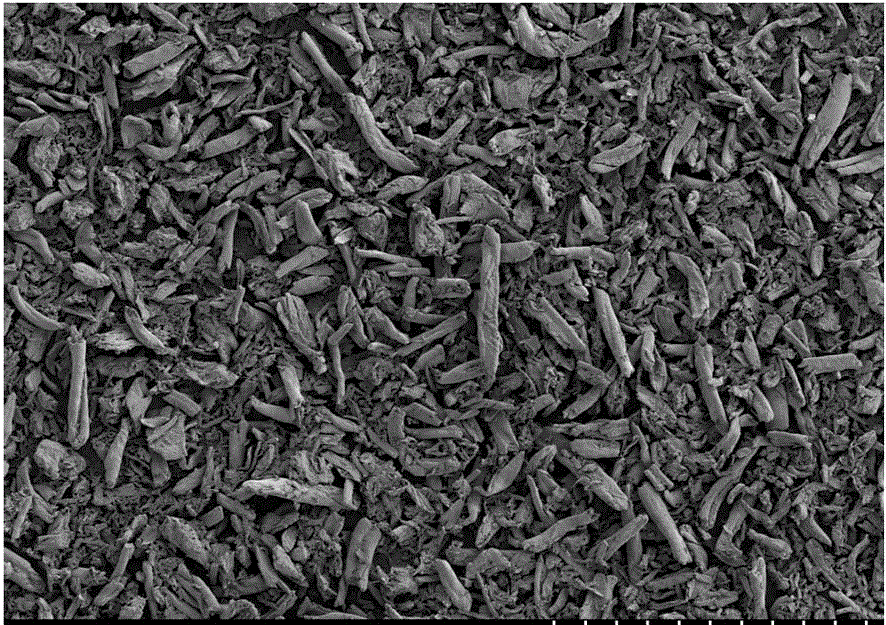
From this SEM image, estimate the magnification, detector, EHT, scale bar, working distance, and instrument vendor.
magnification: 0.15 K X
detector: SE(M)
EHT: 3 kV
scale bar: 300000 nm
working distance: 13.6 mm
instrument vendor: Hitachi